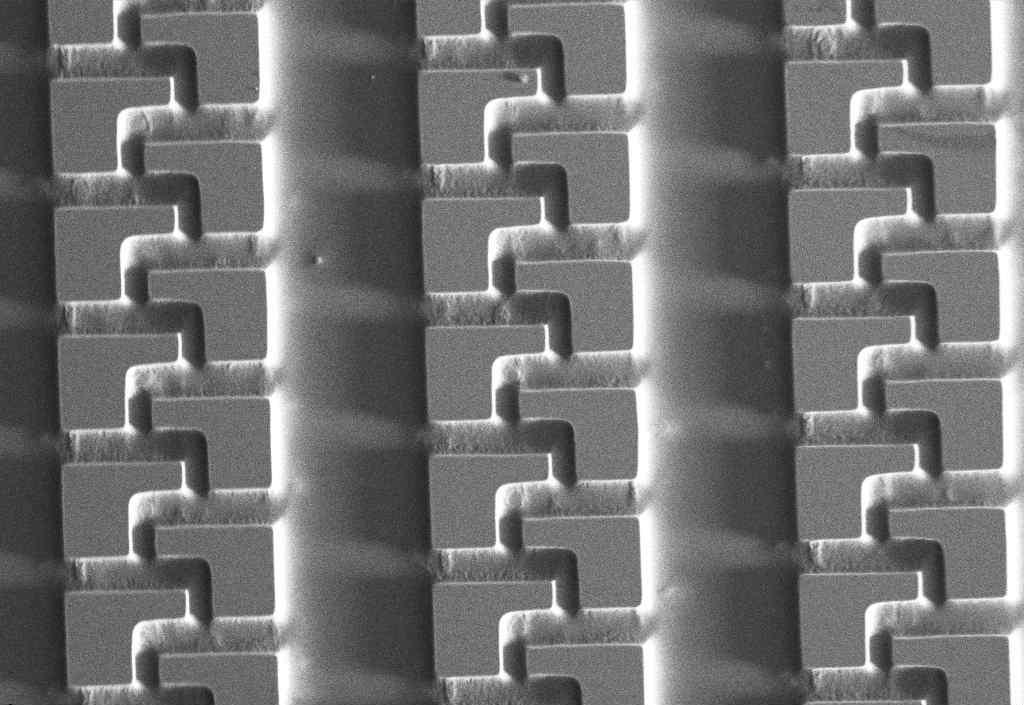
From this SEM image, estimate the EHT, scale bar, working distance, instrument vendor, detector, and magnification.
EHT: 27 kV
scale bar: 20000 nm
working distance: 15 mm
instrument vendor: Zeiss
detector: SE1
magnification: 0.08 K X